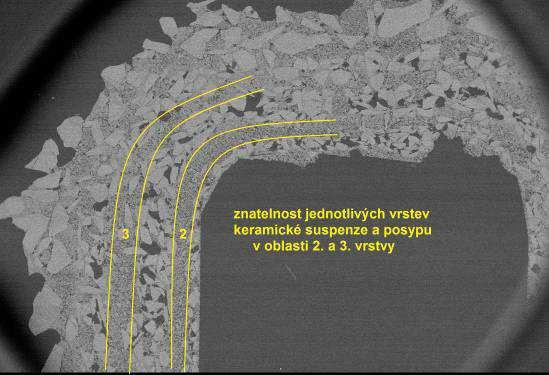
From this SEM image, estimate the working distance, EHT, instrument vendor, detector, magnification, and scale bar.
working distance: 45 mm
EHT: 10 kV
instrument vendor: JEOL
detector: BEC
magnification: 0.01 K X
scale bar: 2000000 nm